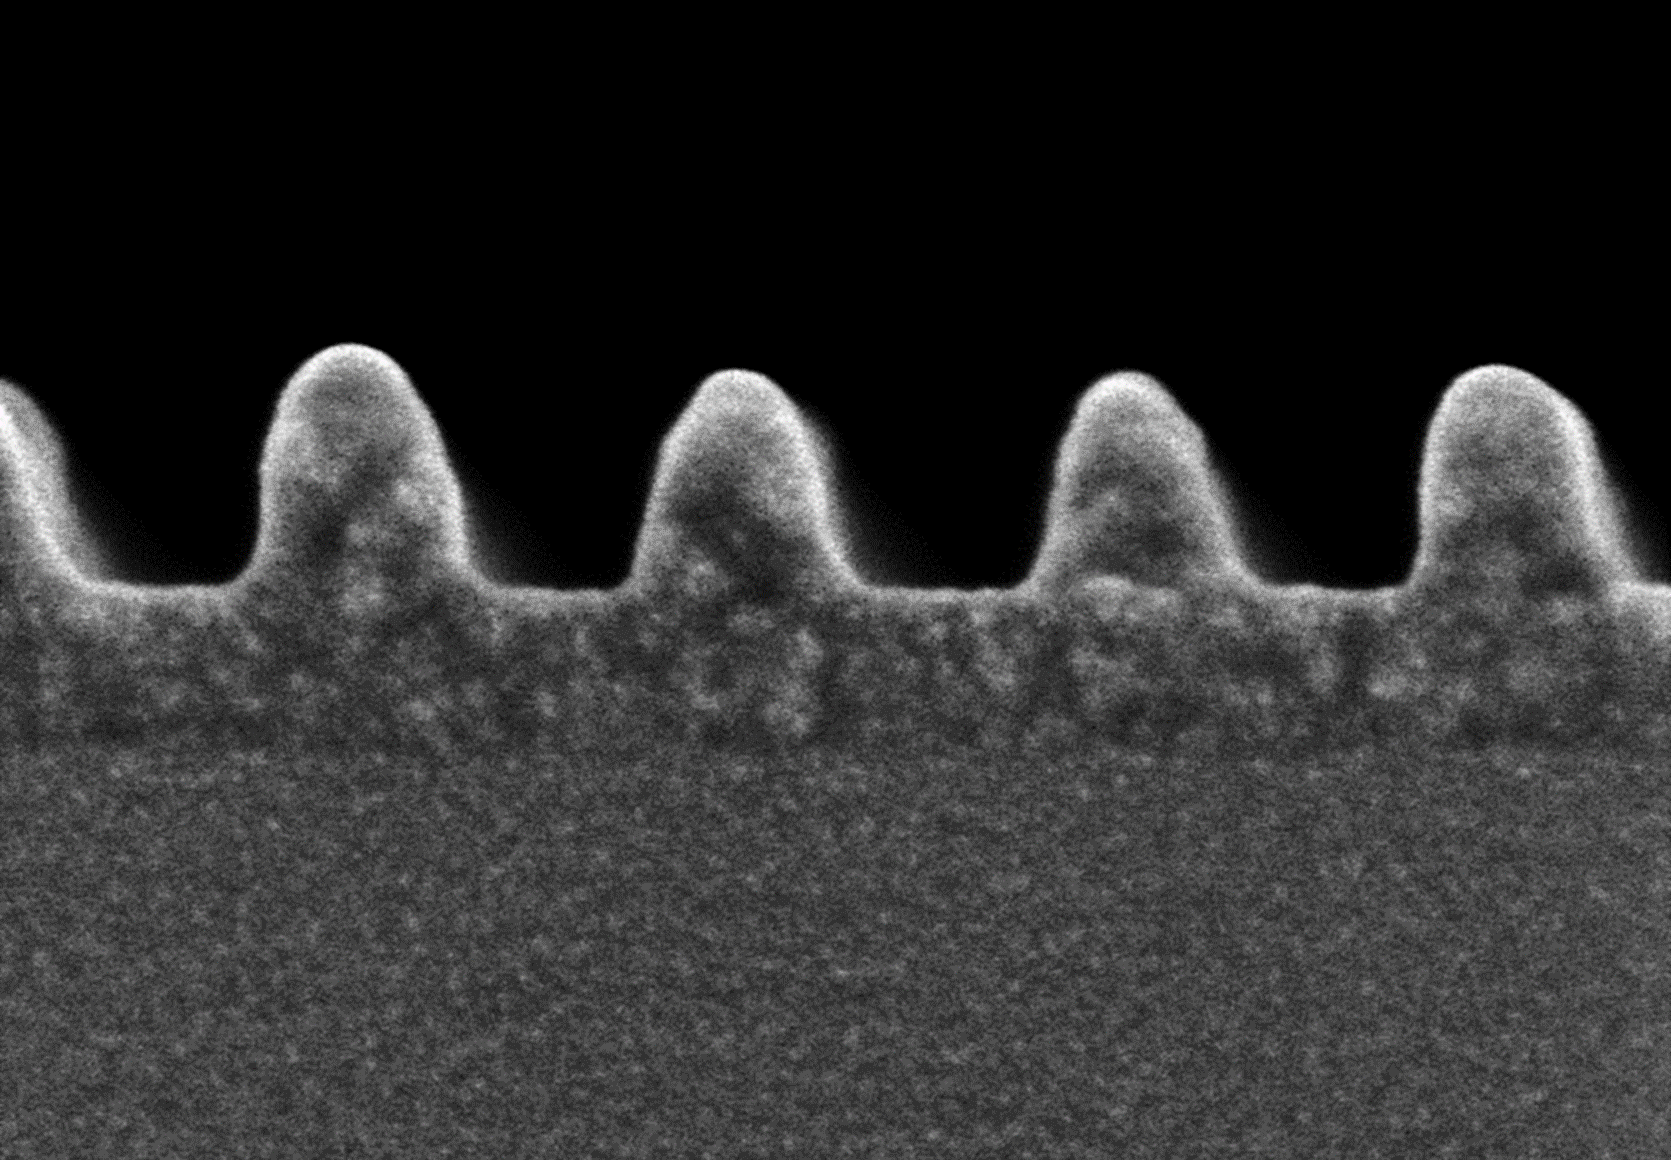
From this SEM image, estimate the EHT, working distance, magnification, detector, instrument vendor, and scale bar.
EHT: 0.5 kV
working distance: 2 mm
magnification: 300 K X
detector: SEU+SET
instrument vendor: Hitachi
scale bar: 100 nm